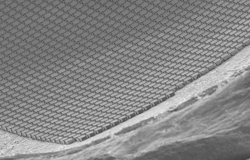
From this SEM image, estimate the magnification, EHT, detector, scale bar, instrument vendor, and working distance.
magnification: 0.05 K X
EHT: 10 kV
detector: SE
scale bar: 1000000 nm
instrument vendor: Hitachi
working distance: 35.7 mm